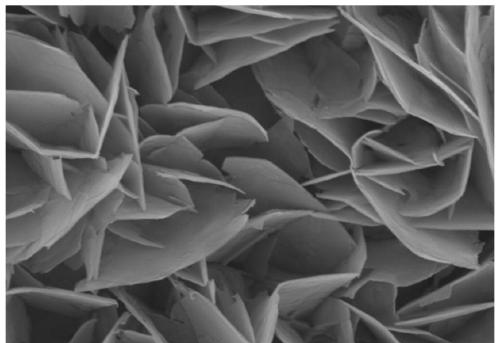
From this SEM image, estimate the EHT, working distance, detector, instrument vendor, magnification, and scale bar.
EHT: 15 kV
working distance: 8.1 mm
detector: SE(U)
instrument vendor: Hitachi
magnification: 20 K X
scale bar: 2000 nm